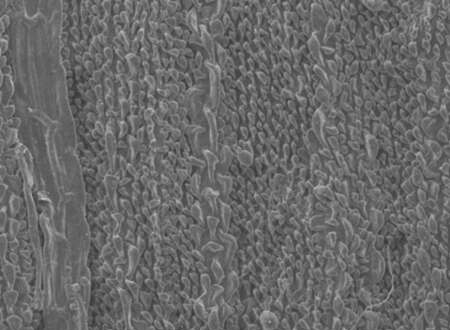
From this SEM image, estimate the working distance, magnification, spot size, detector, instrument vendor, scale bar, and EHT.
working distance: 16.2 mm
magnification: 0.2 K X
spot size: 3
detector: SE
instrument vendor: FEI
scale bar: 100000 nm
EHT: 10 kV